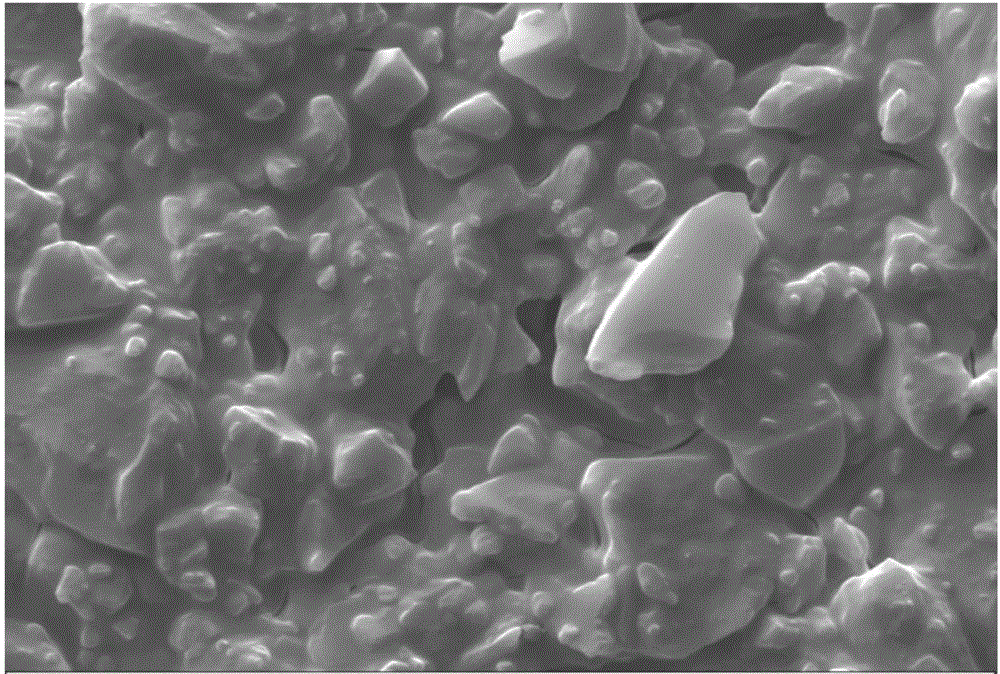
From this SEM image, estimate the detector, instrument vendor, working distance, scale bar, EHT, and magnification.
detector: SE1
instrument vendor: Zeiss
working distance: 9 mm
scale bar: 2000 nm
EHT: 20 kV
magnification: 2 K X